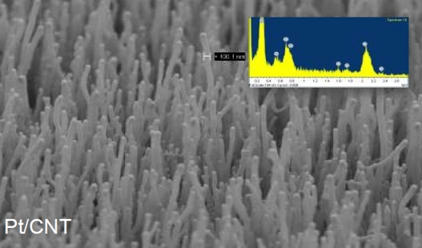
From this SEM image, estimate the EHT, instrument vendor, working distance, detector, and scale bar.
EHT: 5 kV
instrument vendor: Zeiss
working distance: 4 mm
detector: SE2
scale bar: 200 nm